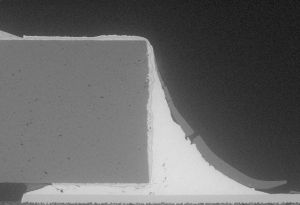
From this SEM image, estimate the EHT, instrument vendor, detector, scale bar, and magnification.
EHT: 5 kV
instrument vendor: Hitachi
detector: BSECOMP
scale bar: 400000 nm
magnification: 0.12 K X